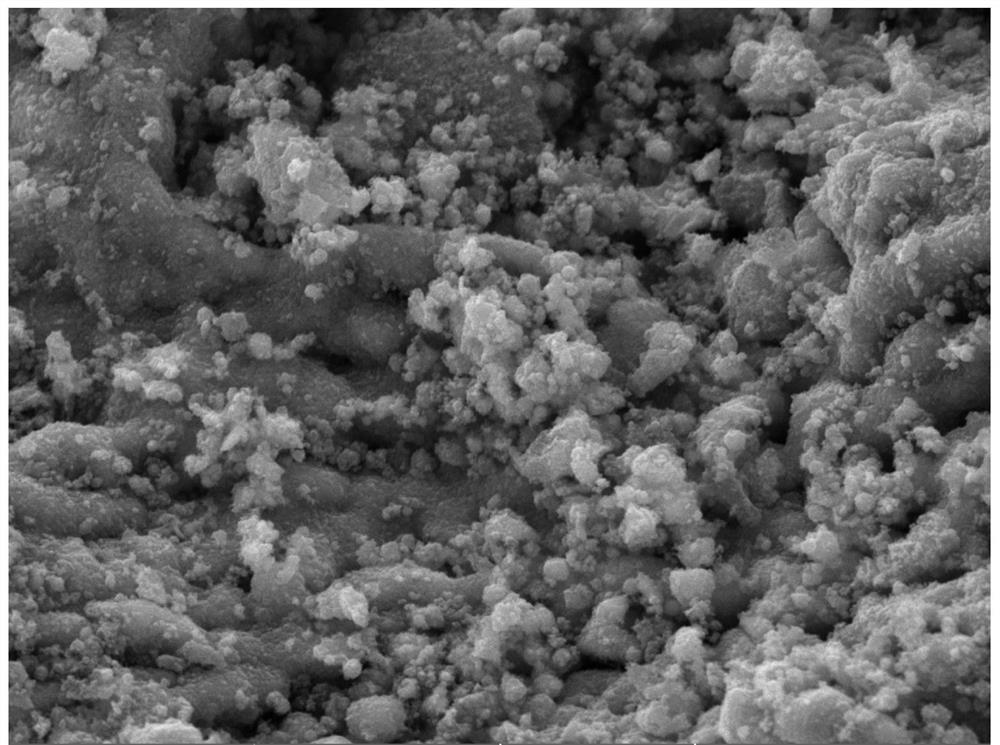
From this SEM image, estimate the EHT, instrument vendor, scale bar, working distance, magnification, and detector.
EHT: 20 kV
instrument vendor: Tescan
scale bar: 5000 nm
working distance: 11.72 mm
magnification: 10 K X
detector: SE